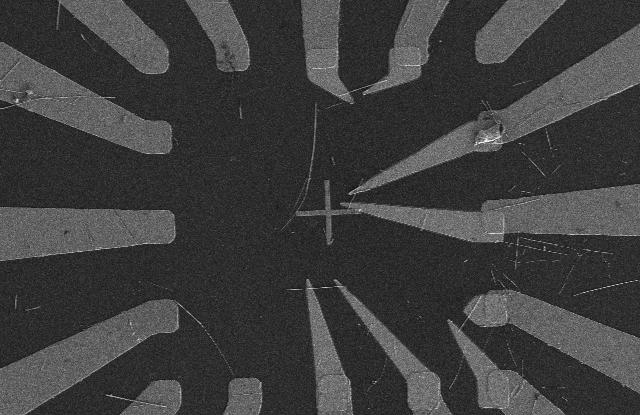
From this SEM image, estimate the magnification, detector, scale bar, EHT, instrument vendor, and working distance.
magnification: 0.901 K X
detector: SE(L)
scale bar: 50000 nm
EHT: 3 kV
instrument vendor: Hitachi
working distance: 7.7 mm